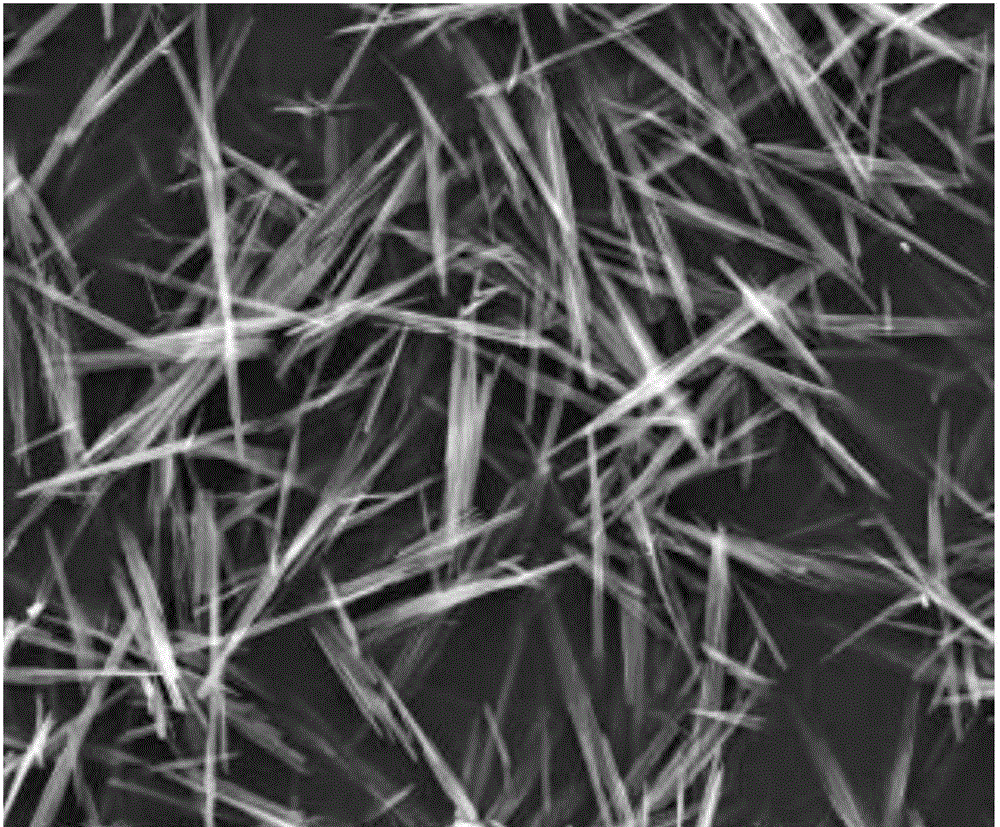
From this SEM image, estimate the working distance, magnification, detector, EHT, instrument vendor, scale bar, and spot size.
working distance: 9.6 mm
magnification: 3 K X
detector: ETD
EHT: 20 kV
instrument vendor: FEI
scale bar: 40000 nm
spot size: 4.5